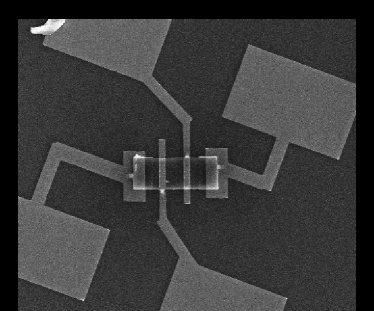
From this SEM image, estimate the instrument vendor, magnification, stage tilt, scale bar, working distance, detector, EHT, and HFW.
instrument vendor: FEI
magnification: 1.714 K X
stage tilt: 0°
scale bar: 20000 nm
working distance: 5.1 mm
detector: ETD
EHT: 15 kV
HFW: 74.7 µm